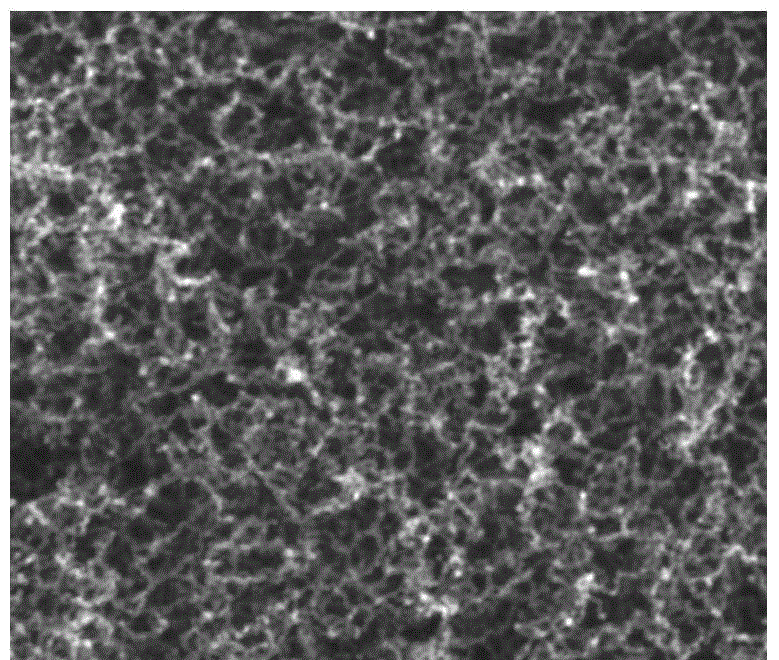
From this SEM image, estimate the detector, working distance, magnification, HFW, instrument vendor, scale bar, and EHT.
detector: TLD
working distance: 5.3 mm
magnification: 100 K X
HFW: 1.28 µm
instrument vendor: FEI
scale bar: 400 nm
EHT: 15 kV